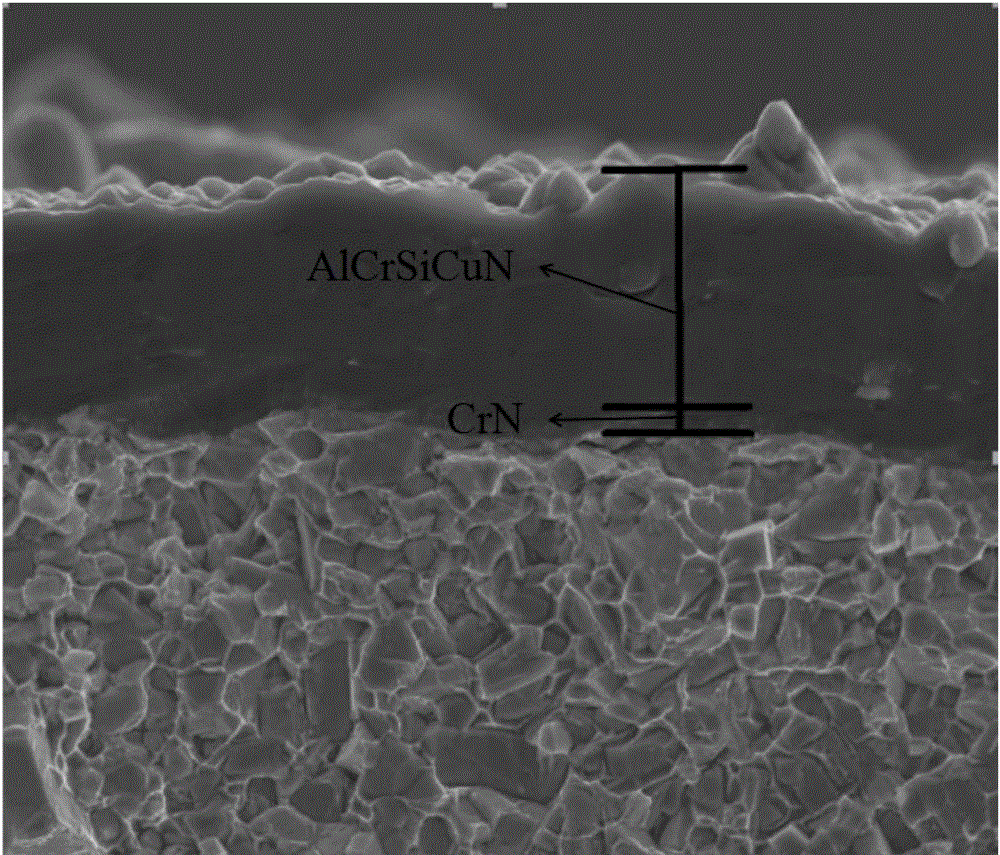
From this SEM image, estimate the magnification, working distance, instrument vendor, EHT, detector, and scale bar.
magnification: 20 K X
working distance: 5.4 mm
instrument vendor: FEI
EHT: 10 kV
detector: ETD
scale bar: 4000 nm